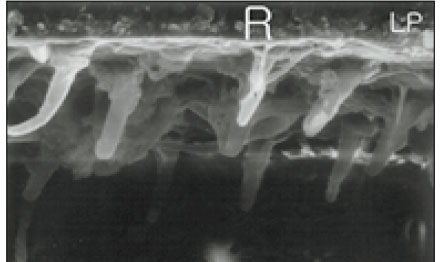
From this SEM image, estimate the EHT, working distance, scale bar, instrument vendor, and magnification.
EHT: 20 kV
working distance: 39 mm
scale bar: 10000 nm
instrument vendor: JEOL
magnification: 3.5 K X